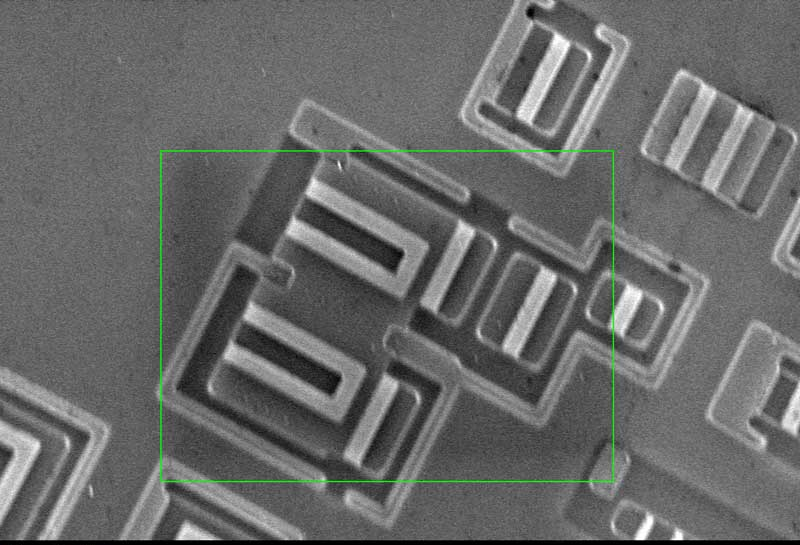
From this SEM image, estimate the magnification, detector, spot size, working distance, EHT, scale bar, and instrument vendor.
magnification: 2 K X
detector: SE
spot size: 12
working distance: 7.7 mm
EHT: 20 kV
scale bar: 10000 nm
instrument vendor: other